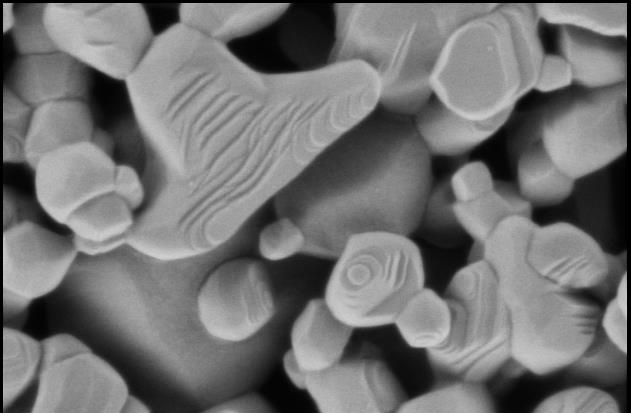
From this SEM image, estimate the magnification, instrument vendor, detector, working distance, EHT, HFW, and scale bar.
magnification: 100 K X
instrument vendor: Thermo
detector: T2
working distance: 3.1 mm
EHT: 0.5 kV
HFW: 1.27 µm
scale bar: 500 nm